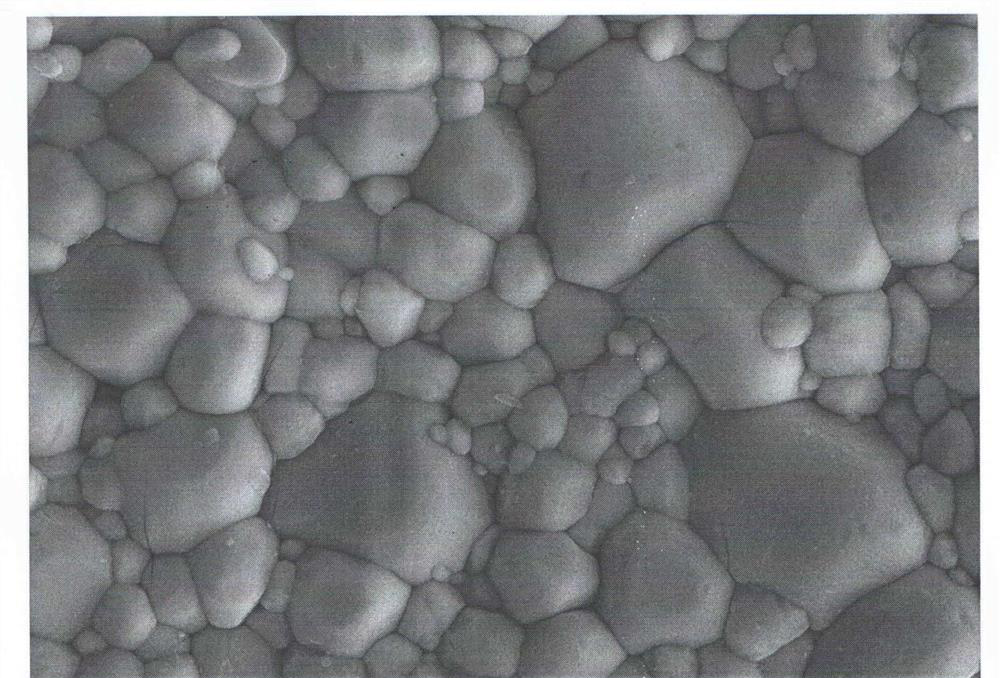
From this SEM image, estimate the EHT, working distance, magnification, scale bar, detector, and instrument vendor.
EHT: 20 kV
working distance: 7 mm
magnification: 3 K X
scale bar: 2000 nm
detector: SE2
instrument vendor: Zeiss